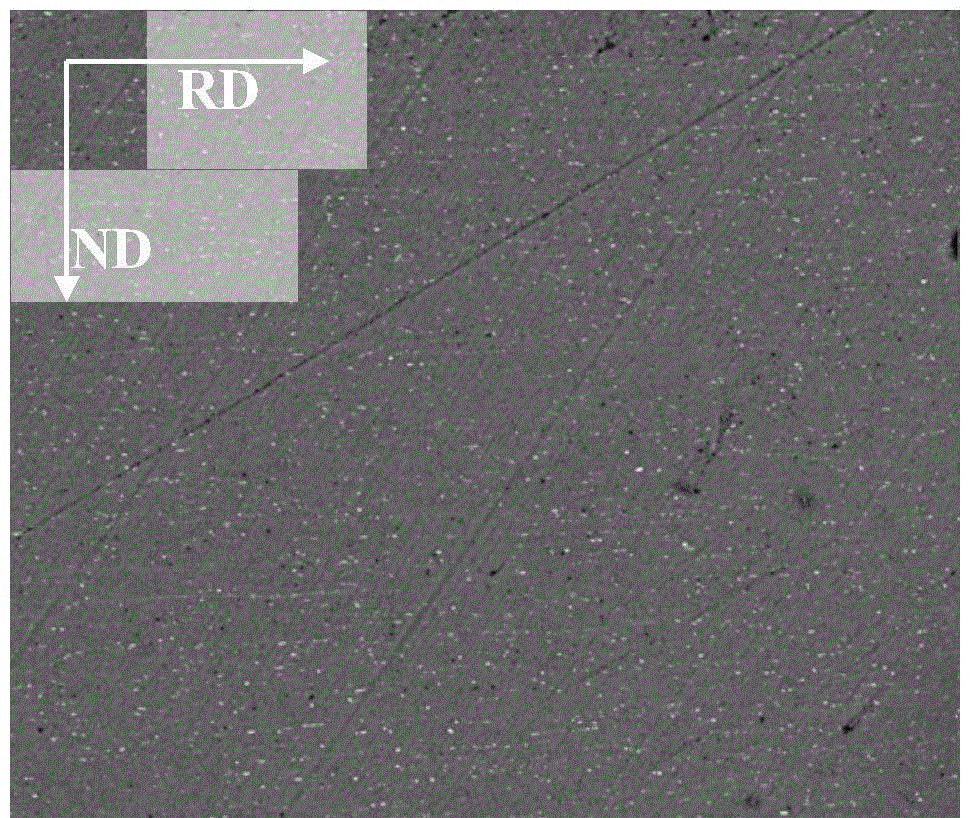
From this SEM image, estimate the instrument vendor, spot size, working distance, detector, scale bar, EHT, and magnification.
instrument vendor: FEI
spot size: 5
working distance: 10.1 mm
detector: DualBSD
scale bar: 100000 nm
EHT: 20 kV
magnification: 1 K X